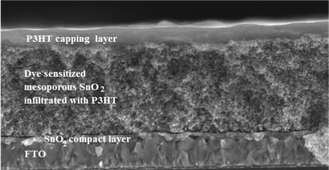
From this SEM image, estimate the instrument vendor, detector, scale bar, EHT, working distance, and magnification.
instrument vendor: JEOL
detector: SE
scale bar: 2500 nm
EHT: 5 kV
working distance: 9.8 mm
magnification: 18 K X